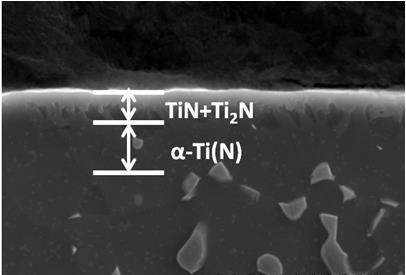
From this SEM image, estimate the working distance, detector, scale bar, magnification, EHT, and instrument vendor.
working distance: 6.7 mm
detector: SE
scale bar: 10000 nm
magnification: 5 K X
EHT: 20 kV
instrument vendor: Hitachi